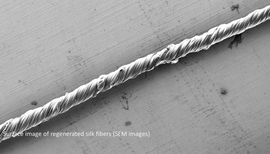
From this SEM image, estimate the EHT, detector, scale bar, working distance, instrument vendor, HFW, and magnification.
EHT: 5 kV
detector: SE2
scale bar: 100000 nm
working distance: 9.1 mm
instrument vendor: Zeiss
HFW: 1751 µm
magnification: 0.172 K X